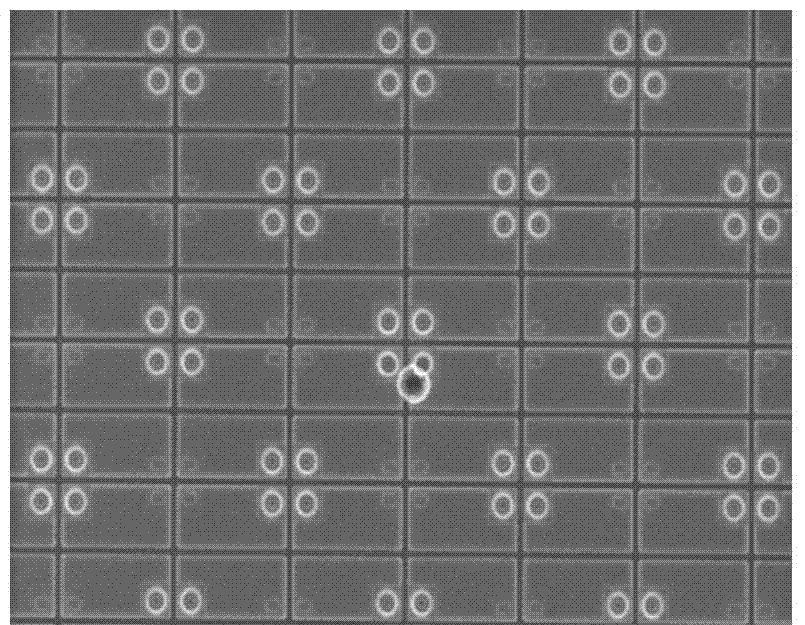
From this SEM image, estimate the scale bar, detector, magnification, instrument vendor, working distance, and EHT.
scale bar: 50000 nm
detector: SE(U)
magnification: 0.8 K X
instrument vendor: Hitachi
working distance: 8 mm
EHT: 15 kV